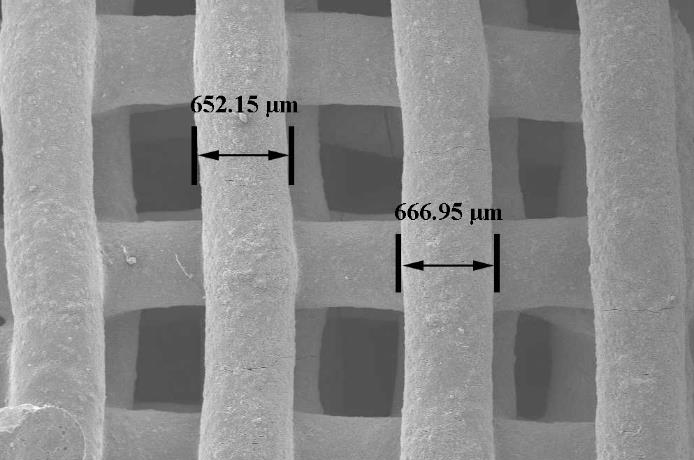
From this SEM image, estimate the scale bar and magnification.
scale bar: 1000000 nm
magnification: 0.02 K X